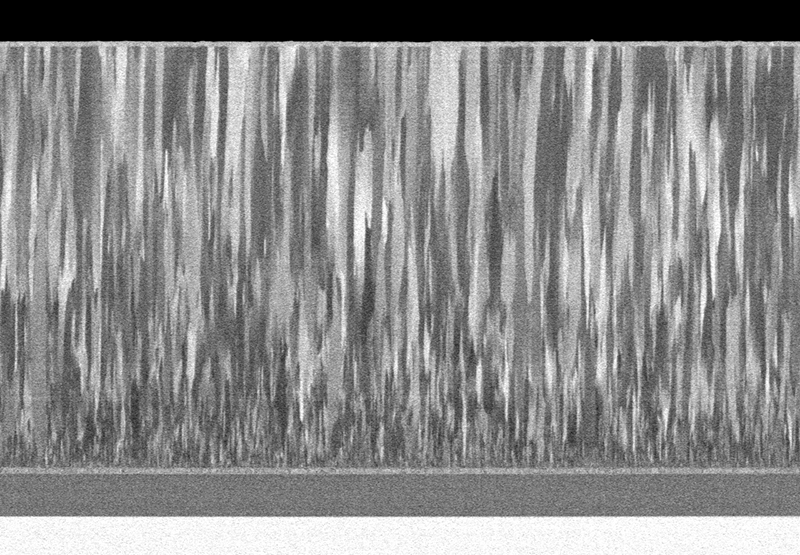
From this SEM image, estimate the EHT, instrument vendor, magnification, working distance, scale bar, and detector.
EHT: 1 kV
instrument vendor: Hitachi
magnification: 7 K X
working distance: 2.9 mm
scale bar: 5000 nm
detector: LA100(U)